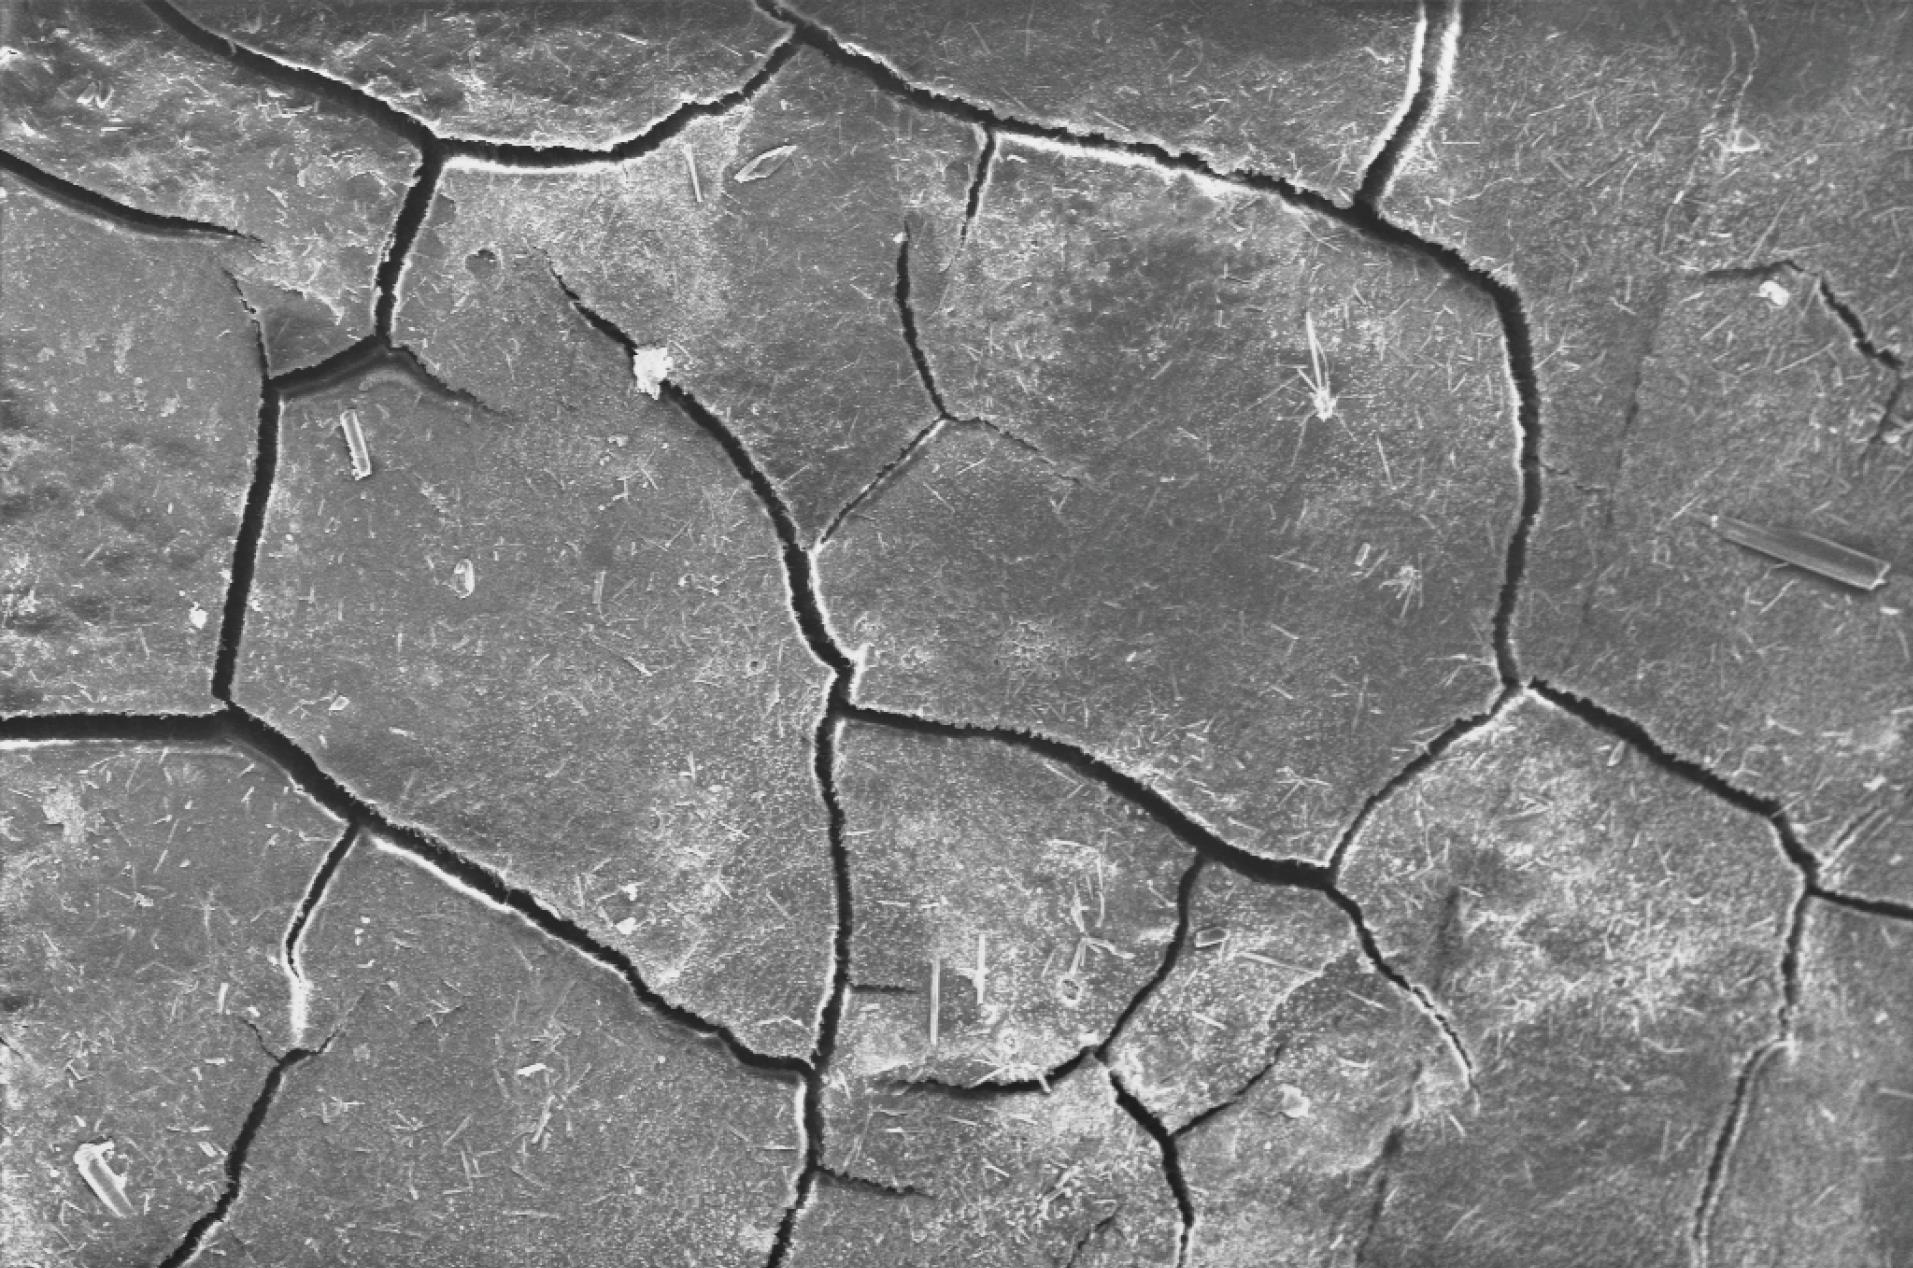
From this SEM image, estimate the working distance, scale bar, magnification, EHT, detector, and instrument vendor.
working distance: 8.9 mm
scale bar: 100000 nm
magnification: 0.1 K X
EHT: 20 kV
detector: SE2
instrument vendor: Zeiss